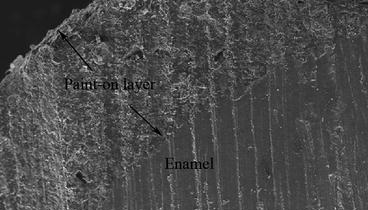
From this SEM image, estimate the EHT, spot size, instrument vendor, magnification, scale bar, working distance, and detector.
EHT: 10 kV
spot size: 4.5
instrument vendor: FEI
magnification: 0.202 K X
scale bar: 100000 nm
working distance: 10.1 mm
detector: SE